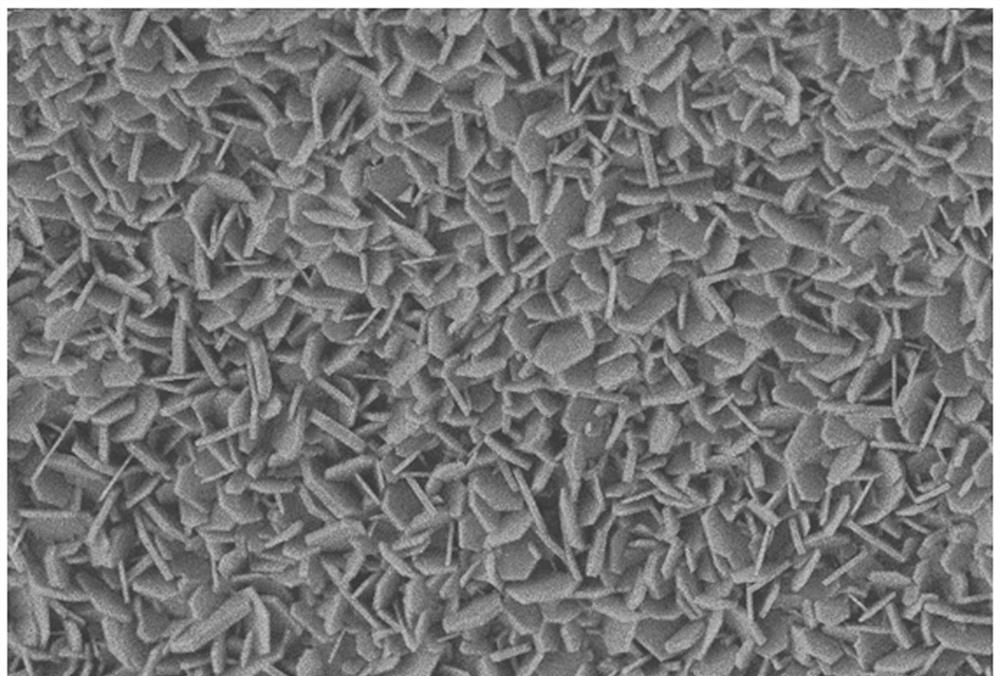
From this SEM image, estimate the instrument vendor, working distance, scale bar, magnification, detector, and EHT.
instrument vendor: Zeiss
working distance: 5.7 mm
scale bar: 2000 nm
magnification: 5 K X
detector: SE2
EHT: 5 kV